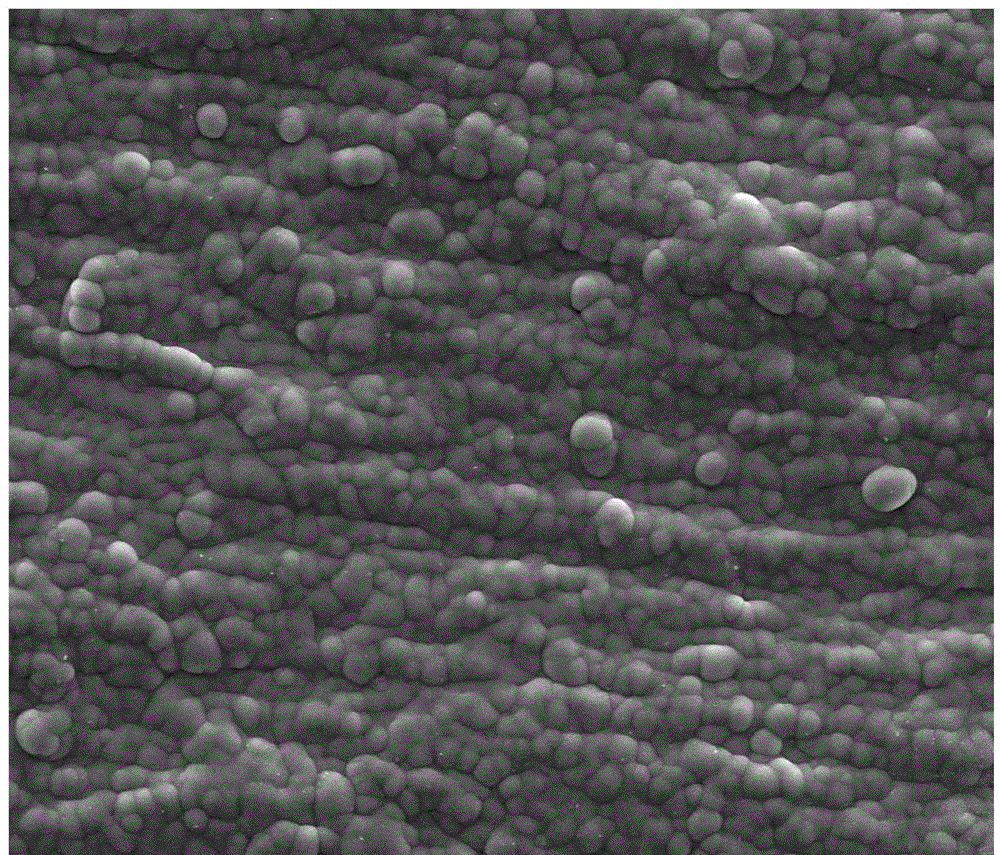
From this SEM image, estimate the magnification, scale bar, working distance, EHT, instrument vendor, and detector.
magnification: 5 K X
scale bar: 20000 nm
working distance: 12.5 mm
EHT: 20 kV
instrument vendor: FEI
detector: SE